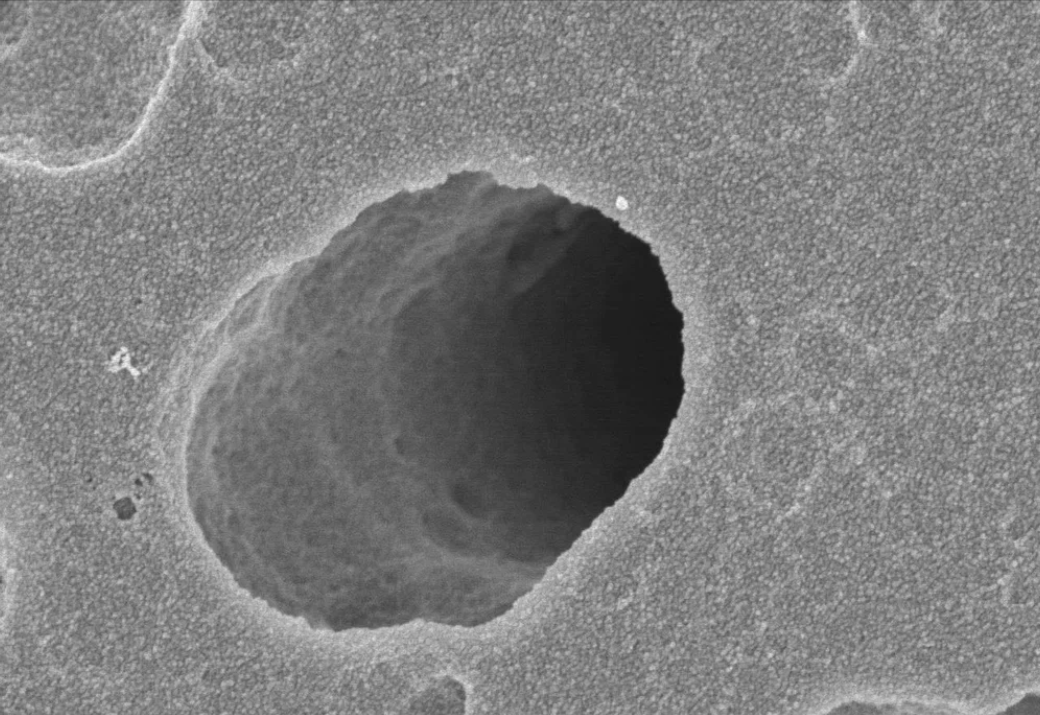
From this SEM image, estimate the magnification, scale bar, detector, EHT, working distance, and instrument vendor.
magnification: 25 K X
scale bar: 2000 nm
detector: SE(U)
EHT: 3 kV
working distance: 6.5 mm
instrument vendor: Hitachi